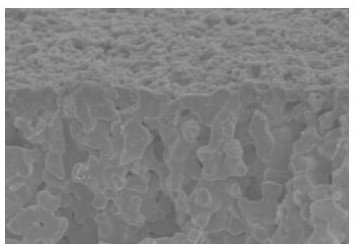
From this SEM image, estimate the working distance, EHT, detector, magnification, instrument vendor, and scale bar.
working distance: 11.1 mm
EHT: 3 kV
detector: SE(M)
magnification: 18 K X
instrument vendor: Hitachi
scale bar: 3000 nm